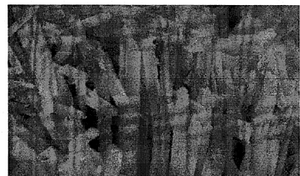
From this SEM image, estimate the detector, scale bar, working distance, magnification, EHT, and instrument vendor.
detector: SE(M)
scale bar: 500 nm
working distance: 7.6 mm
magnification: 100 K X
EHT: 5 kV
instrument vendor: Hitachi